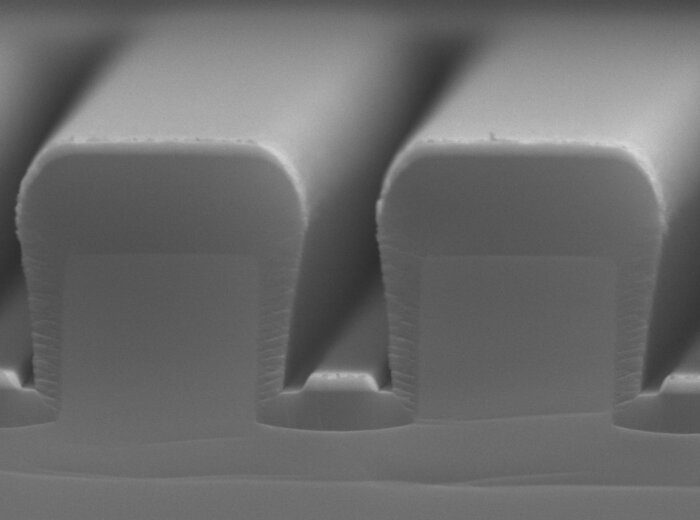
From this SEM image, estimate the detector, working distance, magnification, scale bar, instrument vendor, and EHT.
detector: LED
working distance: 5.6 mm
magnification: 30 K X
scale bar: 100 nm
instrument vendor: JEOL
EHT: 15 kV